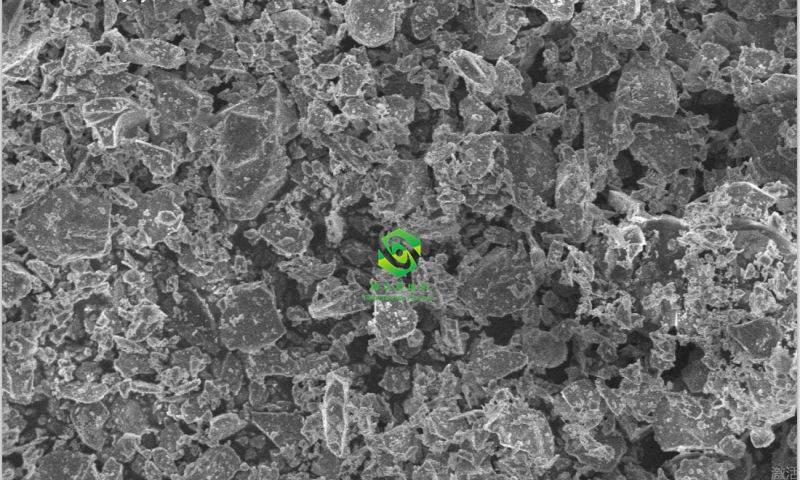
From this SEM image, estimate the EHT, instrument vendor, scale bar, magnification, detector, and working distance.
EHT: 5 kV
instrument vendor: Hitachi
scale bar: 20000 nm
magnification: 2 K X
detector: SE(U)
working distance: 8.2 mm